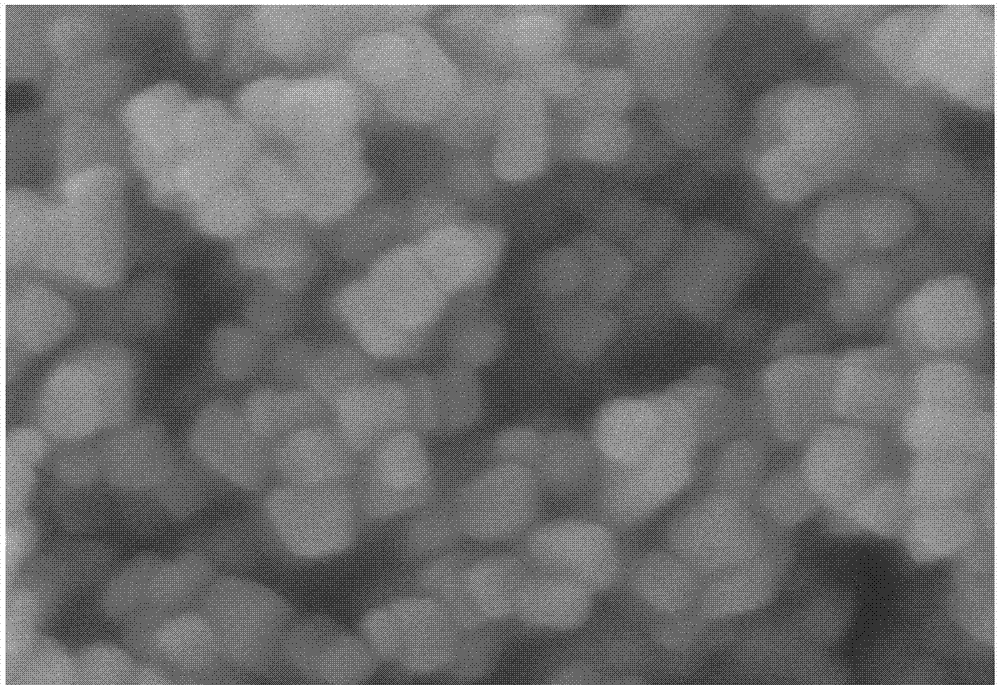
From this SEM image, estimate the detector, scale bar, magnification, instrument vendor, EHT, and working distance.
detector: SE(UL)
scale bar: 200 nm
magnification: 200 K X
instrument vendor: Hitachi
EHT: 5 kV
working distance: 8.2 mm